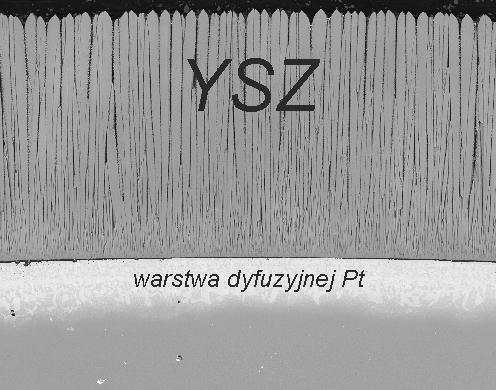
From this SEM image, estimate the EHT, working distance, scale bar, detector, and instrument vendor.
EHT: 15 kV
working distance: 9.4 mm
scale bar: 200000 nm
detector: BSED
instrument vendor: FEI